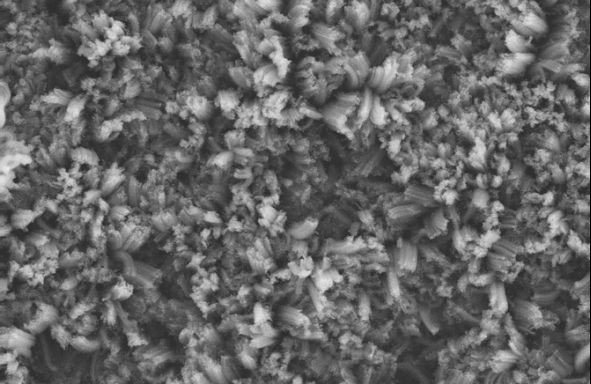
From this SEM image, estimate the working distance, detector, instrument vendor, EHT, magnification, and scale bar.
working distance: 6 mm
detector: SE2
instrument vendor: Zeiss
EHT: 10 kV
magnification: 0.5 K X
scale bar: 20000 nm